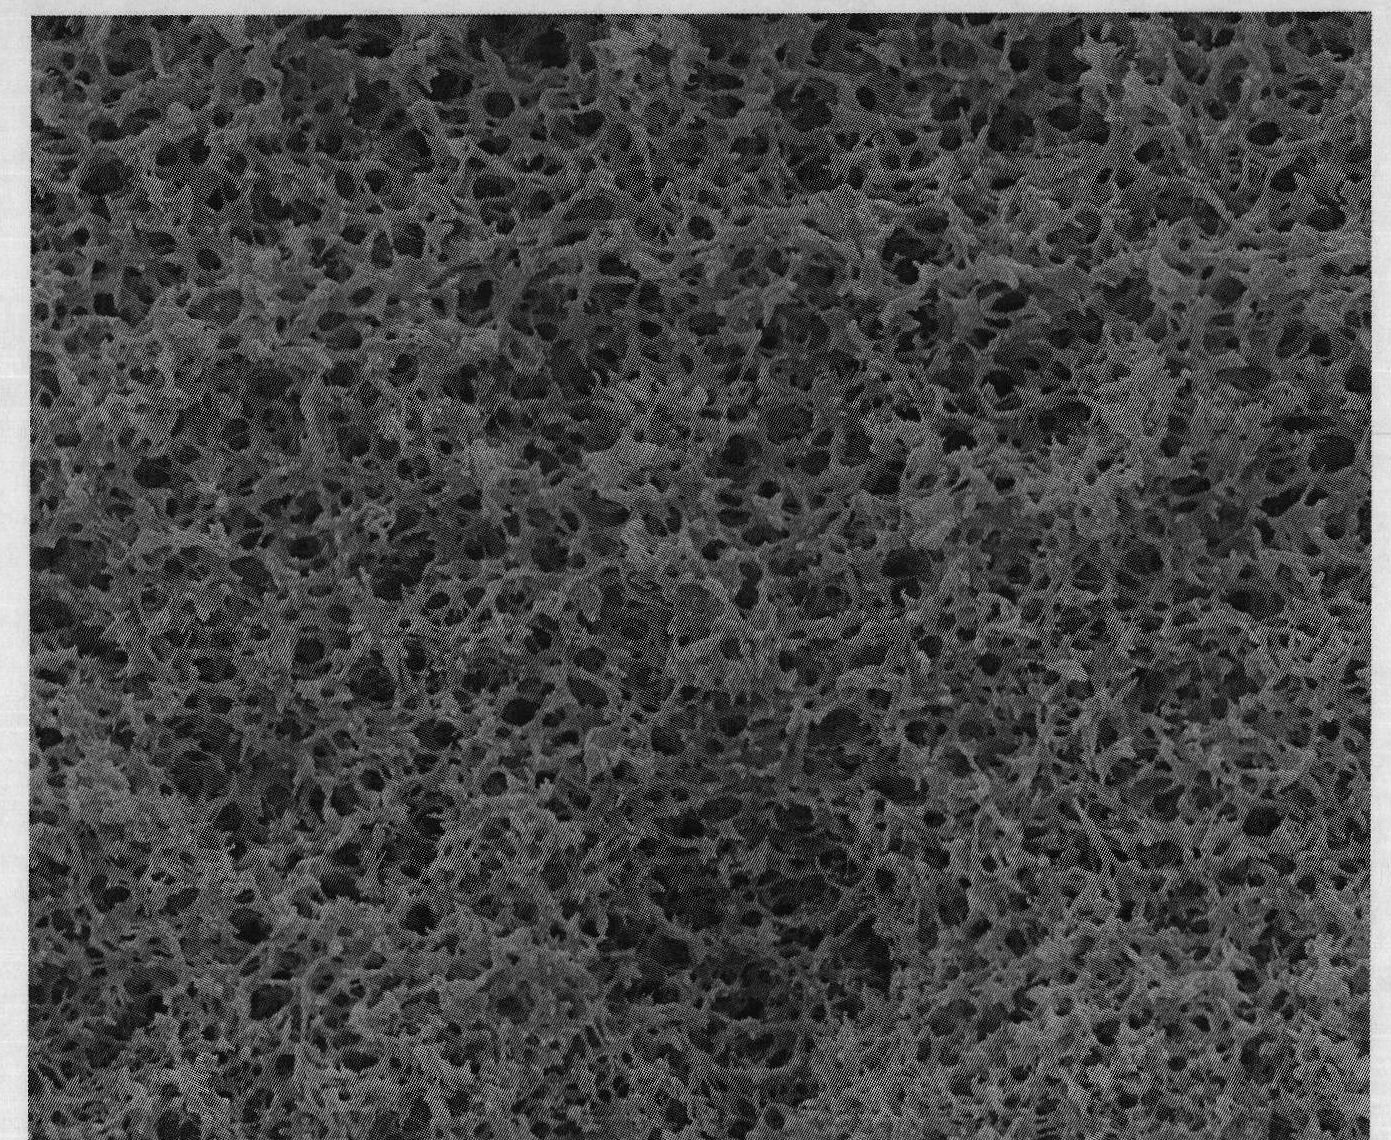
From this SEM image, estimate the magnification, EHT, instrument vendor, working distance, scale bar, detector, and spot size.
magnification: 10 K X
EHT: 15 kV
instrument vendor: FEI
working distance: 10 mm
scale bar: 5000 nm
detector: ETD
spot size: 4